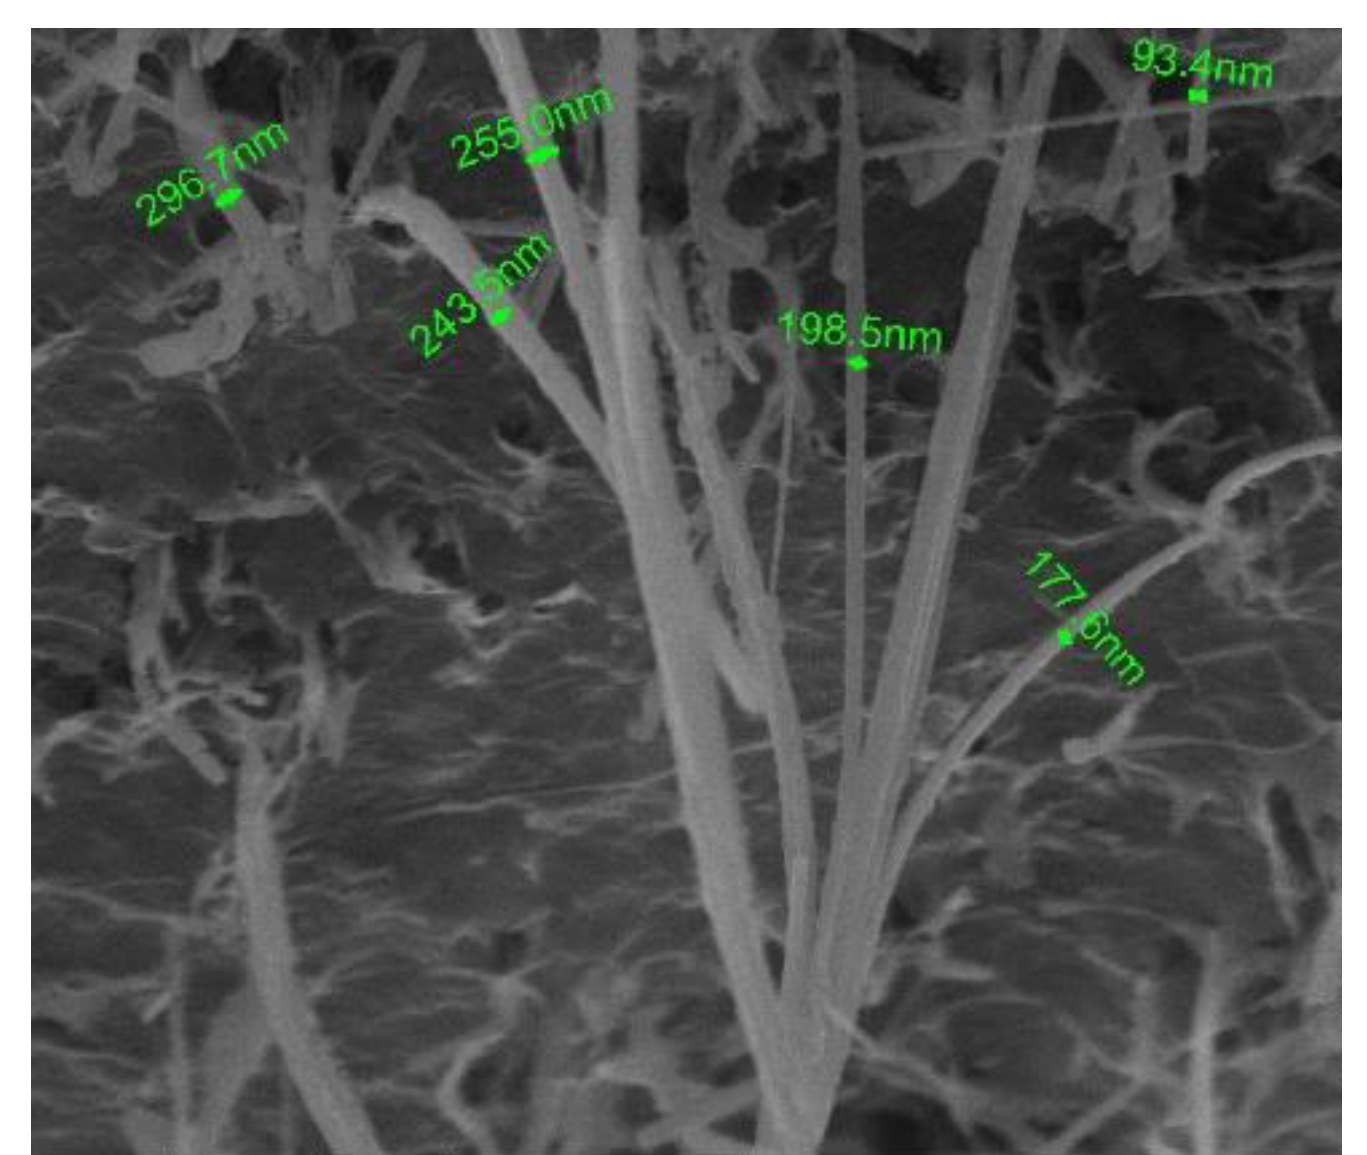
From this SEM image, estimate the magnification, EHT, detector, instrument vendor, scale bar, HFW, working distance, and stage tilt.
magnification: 20 K X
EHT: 20 kV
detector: SE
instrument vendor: FEI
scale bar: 5000 nm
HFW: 13.5 µm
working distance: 9.9 mm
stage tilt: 1°